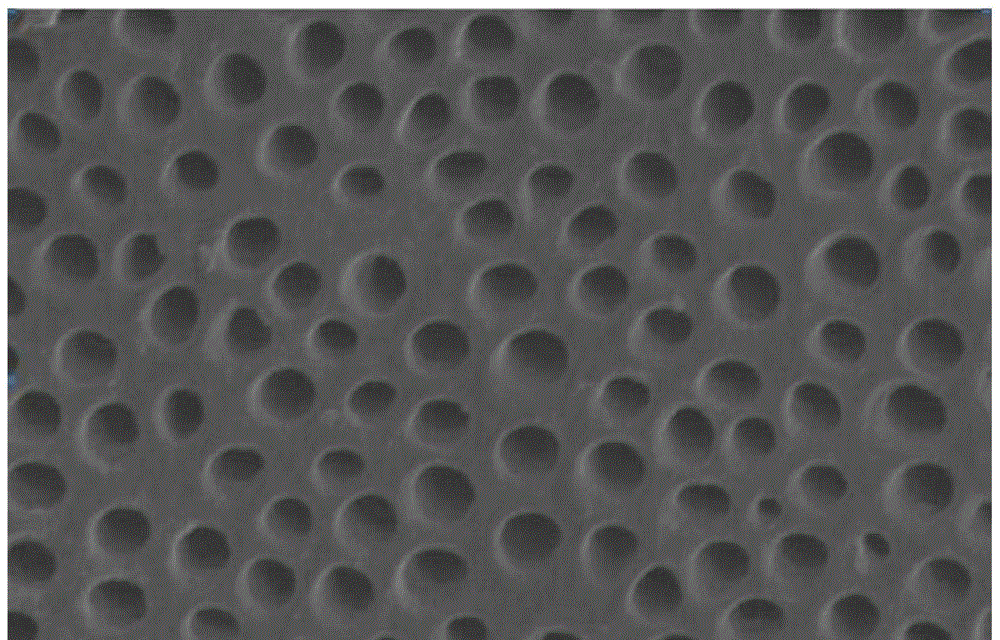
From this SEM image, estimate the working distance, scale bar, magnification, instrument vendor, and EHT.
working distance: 12.2 mm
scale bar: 20000 nm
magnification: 2 K X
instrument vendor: Hitachi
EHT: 5 kV